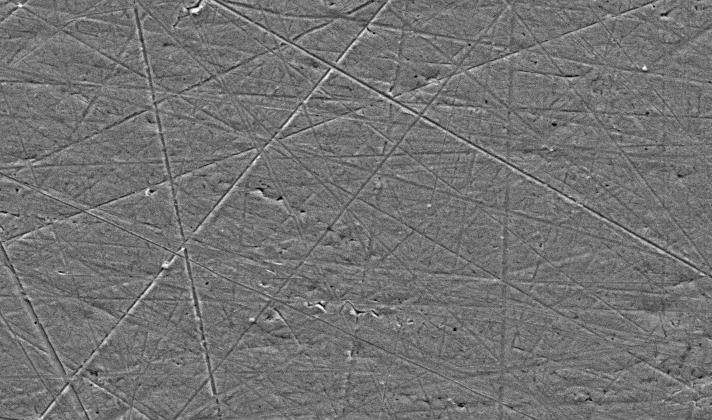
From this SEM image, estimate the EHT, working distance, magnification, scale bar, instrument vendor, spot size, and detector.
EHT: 20 kV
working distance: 11.9 mm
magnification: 1 K X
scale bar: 20000 nm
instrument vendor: FEI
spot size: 5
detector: SE